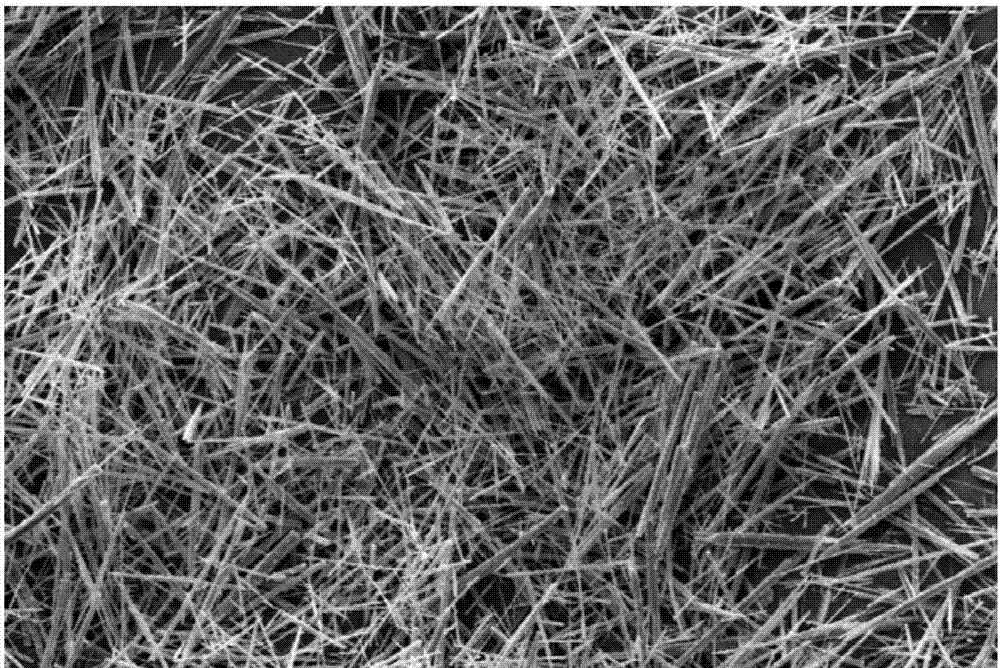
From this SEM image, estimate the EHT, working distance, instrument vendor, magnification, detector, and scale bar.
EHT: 20 kV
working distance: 11 mm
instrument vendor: Zeiss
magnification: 0.177 K X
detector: SE1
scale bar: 100000 nm